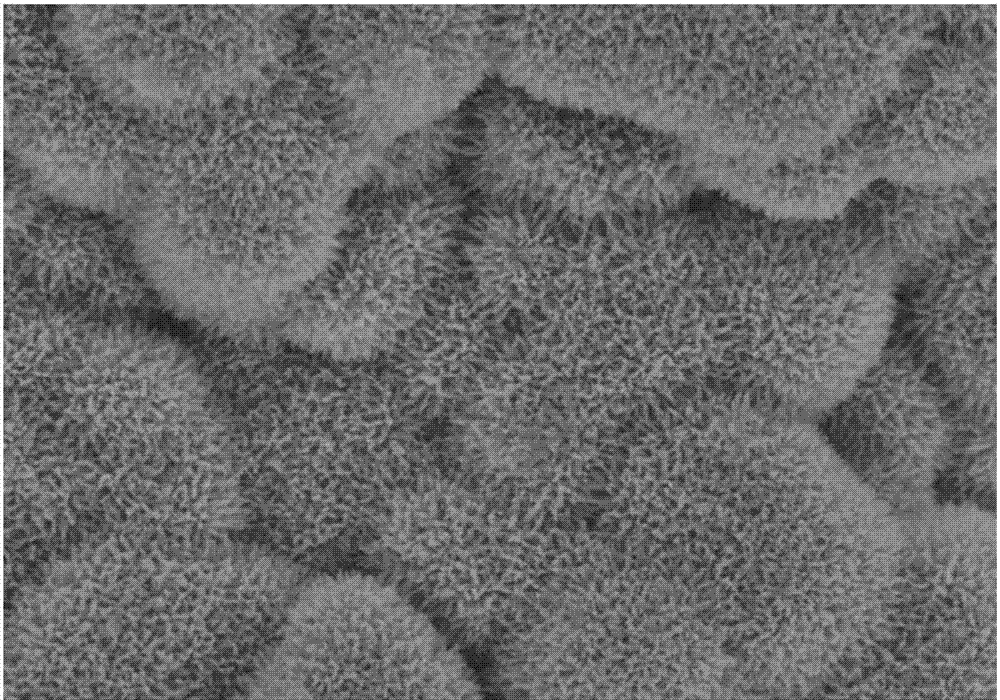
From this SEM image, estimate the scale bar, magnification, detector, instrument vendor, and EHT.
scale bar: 5000 nm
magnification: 10 K X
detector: SE(U)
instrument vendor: Hitachi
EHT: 8 kV